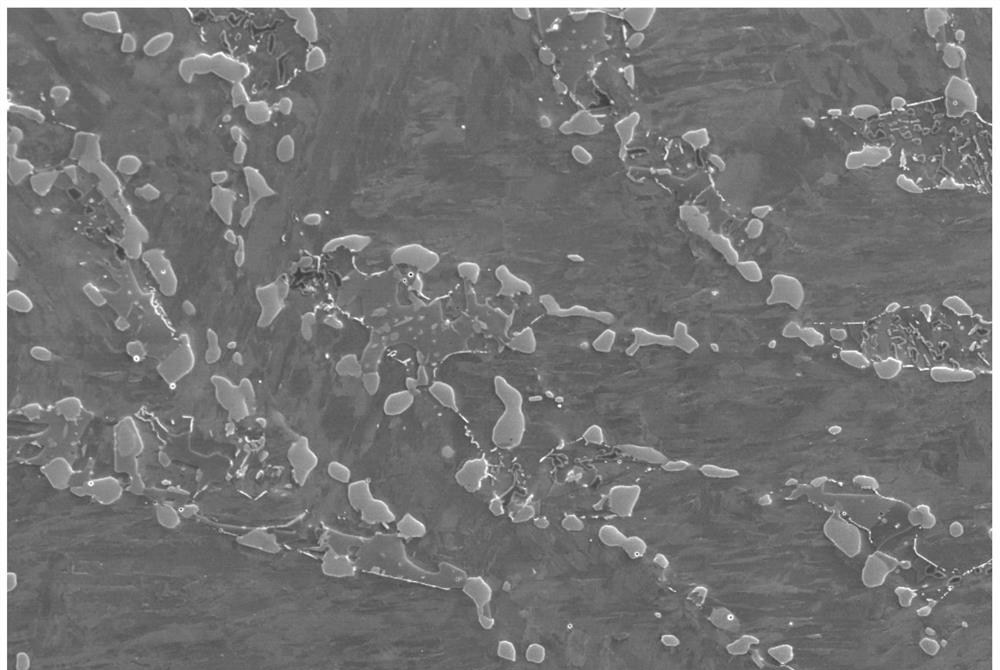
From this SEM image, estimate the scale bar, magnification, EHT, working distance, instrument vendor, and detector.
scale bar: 2000 nm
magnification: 3 K X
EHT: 5 kV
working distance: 8.8 mm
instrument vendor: Zeiss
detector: InLens